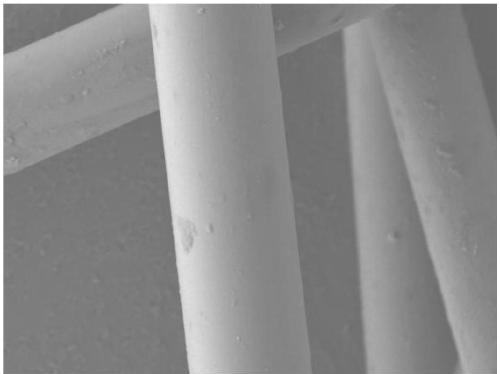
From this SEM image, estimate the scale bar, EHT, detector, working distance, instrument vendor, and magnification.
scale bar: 10000 nm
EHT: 5 kV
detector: LEI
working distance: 6.5 mm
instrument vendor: JEOL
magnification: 2 K X